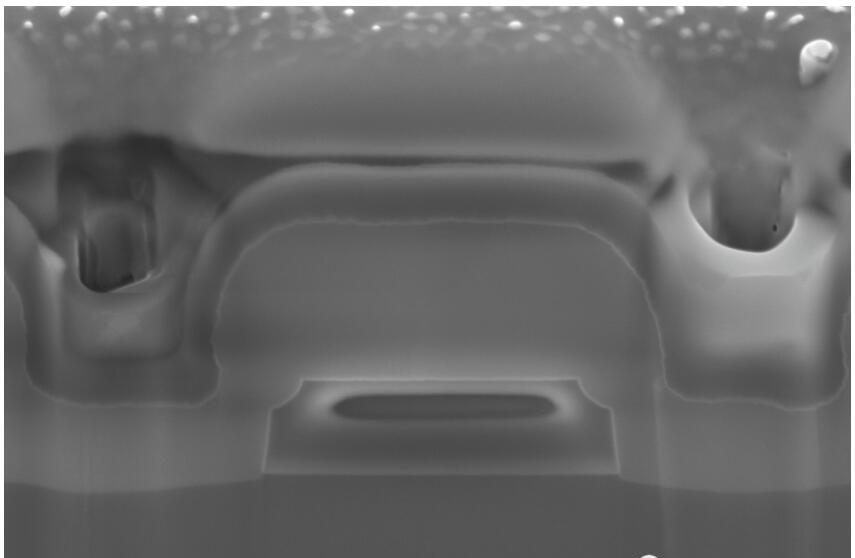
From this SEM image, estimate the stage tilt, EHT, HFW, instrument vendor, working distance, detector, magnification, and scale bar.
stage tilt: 52°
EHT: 5 kV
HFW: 5.08 µm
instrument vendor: FEI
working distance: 4 mm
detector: TLD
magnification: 25 K X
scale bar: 500 nm